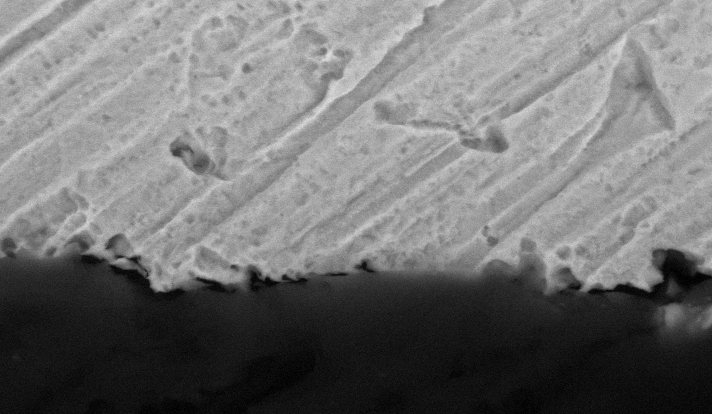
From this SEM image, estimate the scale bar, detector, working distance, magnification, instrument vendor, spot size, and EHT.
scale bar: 10000 nm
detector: BSE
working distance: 10 mm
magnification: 3 K X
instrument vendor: FEI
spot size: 4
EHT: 20 kV